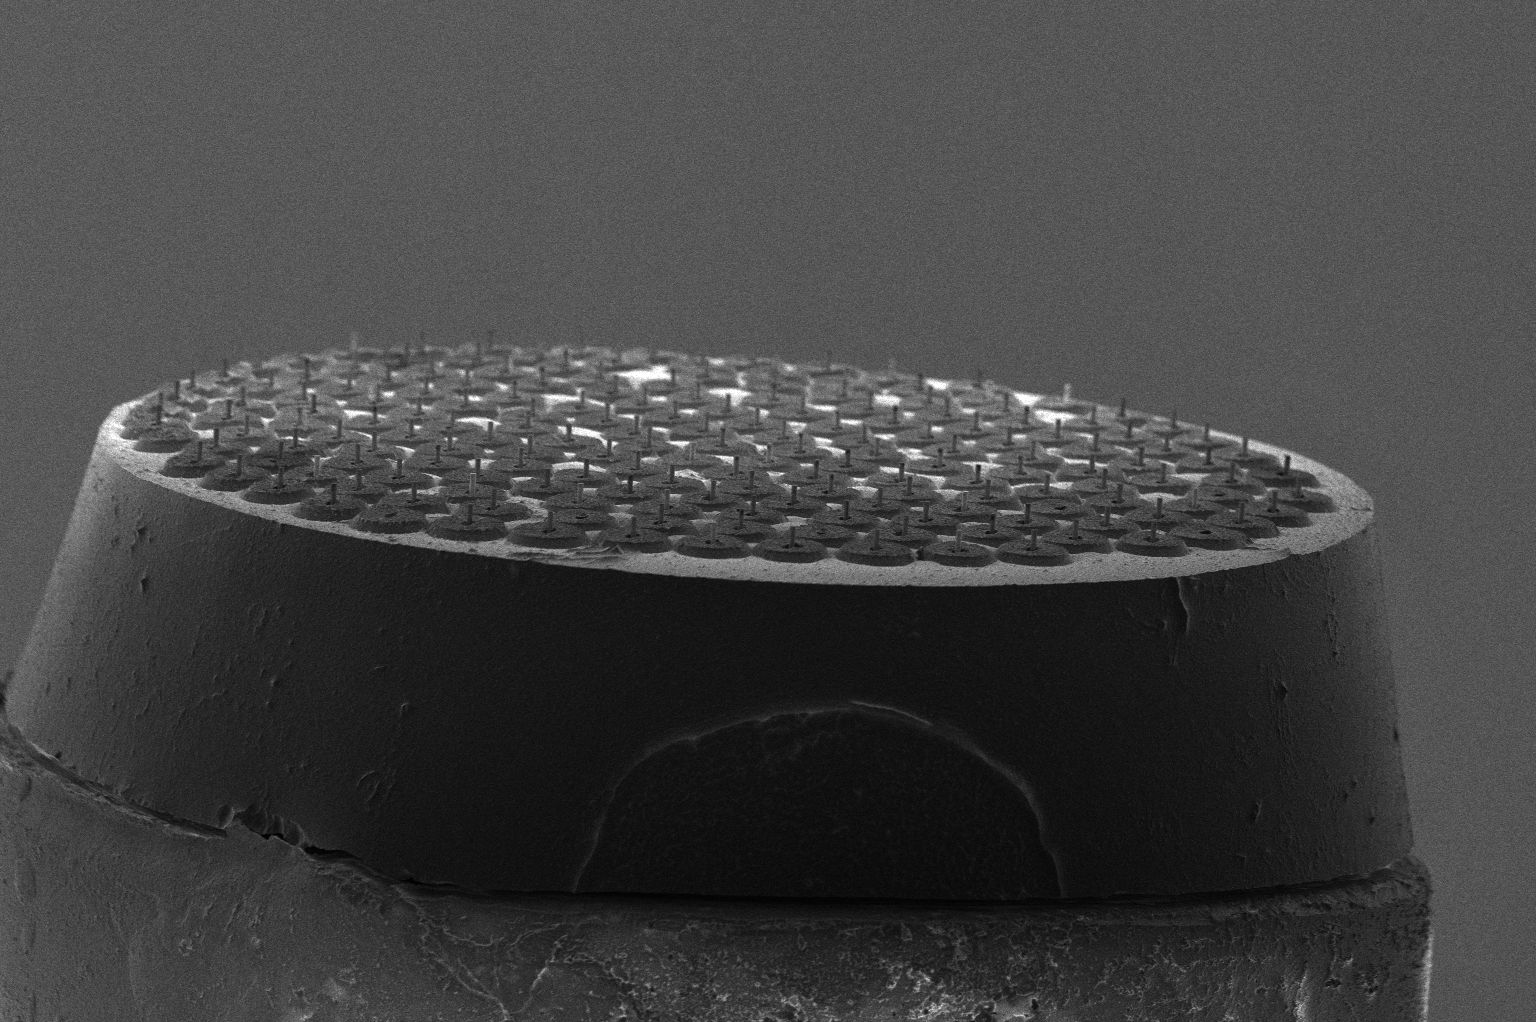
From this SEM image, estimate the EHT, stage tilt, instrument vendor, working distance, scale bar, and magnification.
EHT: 2 kV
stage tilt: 38°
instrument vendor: FEI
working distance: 9.4 mm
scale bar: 500000 nm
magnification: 0.09 K X